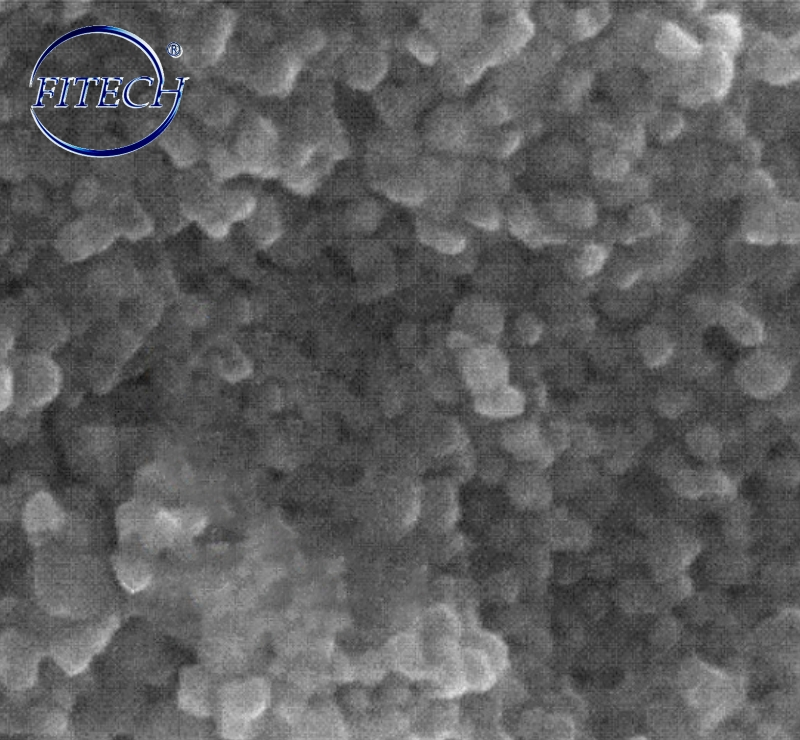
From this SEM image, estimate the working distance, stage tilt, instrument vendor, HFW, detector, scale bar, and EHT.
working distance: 3 mm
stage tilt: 0°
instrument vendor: FEI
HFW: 0.635 µm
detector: TLD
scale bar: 200 nm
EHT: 5 kV